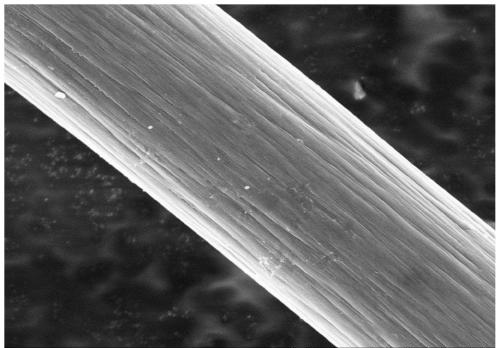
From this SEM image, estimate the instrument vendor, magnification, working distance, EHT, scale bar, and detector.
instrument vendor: Hitachi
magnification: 5 K X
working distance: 10.5 mm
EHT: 5 kV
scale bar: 10000 nm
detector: SE(M)